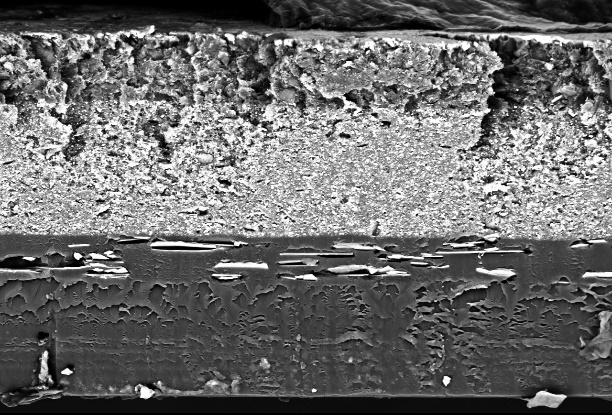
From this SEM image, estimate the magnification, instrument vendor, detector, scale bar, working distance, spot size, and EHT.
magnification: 1 K X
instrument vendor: other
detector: BSE-COMPO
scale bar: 10000 nm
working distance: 12.9 mm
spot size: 16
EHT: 15 kV